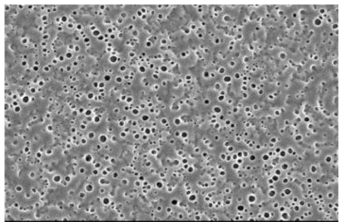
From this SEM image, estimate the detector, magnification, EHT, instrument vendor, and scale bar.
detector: SEI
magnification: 1 K X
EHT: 10 kV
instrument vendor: JEOL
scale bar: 10000 nm